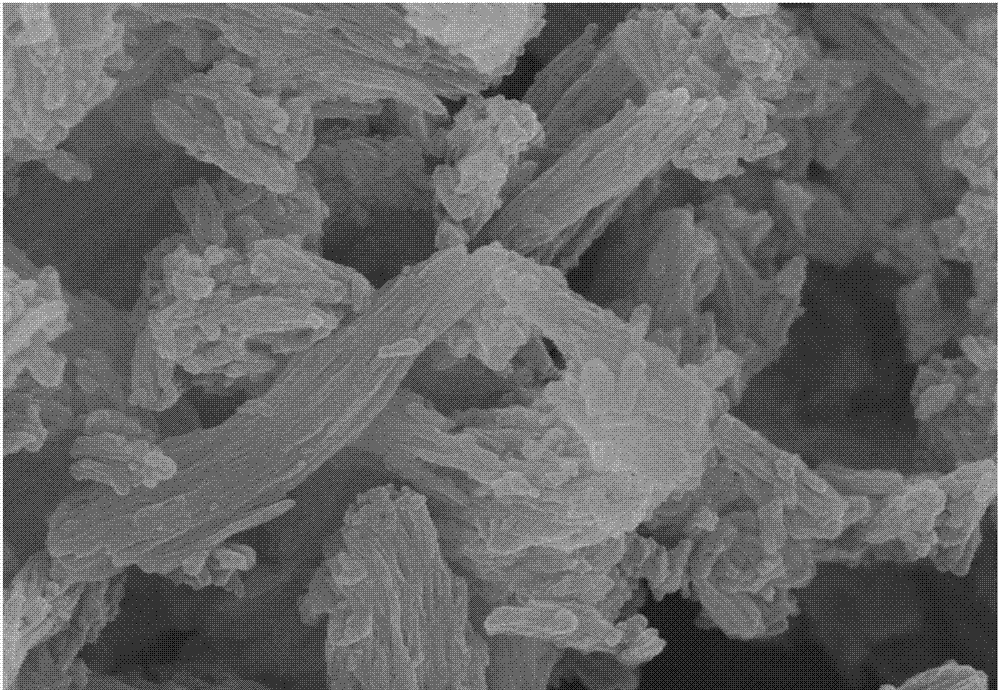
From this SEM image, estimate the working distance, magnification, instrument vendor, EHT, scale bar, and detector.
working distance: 6.1 mm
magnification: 40 K X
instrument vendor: Hitachi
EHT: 10 kV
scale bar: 1000 nm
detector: SE(U)